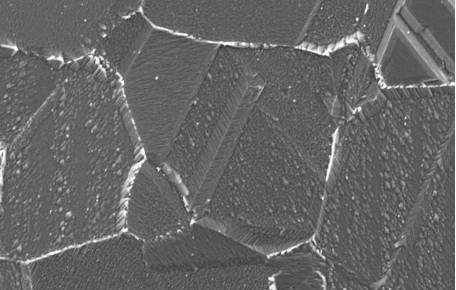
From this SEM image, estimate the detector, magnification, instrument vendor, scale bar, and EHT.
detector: SEI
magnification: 1 K X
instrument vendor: other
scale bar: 20000 nm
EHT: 15 kV